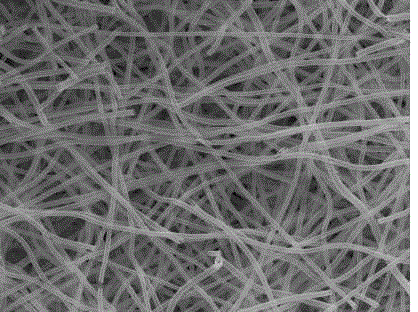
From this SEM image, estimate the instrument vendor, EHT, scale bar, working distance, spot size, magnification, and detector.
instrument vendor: FEI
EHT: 20 kV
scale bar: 10000 nm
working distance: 10.5 mm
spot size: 3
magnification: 10 K X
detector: ETD